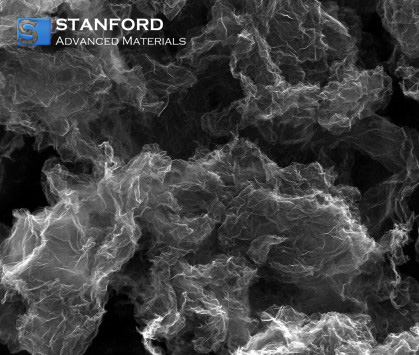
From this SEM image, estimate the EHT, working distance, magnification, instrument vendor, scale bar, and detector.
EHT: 20 kV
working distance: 14.1 mm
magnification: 10 K X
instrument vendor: FEI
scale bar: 10000 nm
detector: SE